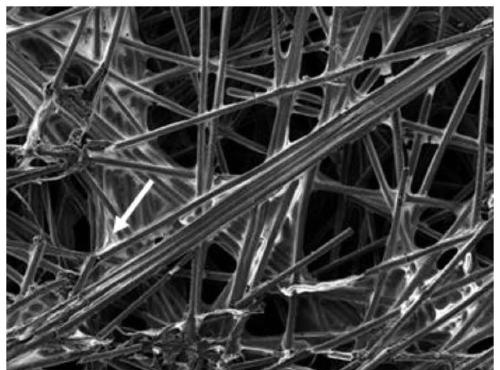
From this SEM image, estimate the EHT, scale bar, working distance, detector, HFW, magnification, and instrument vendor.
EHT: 5 kV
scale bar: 100000 nm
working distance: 9.44 mm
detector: SE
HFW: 554 µm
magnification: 0.5 K X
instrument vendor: Tescan